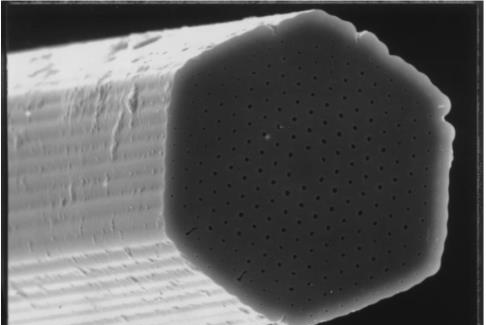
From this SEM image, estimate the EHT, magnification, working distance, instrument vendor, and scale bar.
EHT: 20 kV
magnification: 1.8 K X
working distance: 36 mm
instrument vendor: JEOL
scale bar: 10000 nm